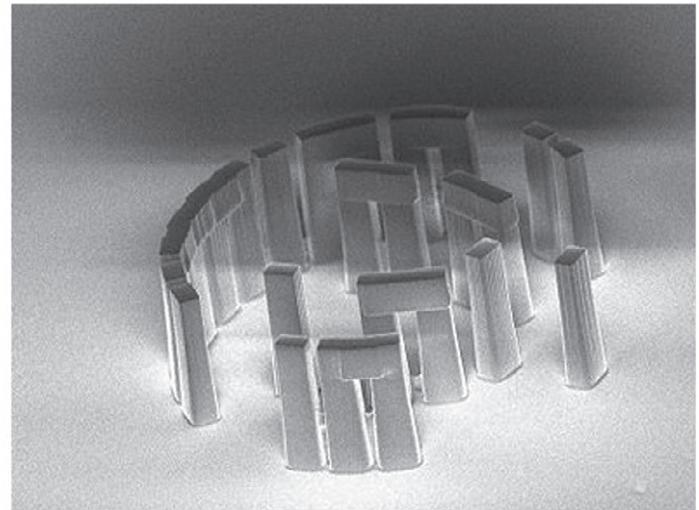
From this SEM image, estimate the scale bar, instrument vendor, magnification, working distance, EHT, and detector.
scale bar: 10000 nm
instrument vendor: JEOL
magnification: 0.85 K X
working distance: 12 mm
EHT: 1 kV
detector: SEI